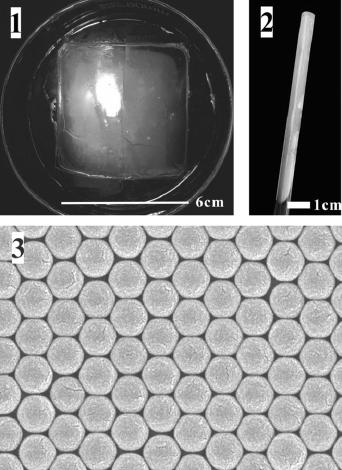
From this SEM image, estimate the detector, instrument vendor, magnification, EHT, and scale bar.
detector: SEI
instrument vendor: JEOL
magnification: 50 K X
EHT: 15 kV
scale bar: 100 nm